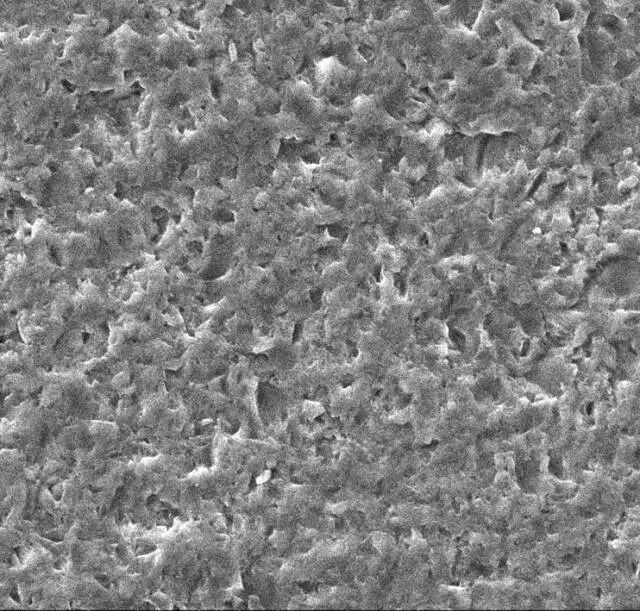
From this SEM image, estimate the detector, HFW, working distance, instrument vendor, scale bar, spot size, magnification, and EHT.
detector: ETD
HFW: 140 µm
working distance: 14.5 mm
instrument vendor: FEI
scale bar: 20000 nm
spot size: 5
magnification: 1 K X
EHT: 20 kV